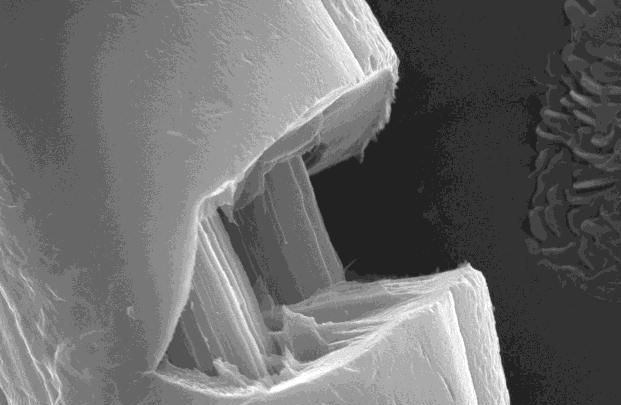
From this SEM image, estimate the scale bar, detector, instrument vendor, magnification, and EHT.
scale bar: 2000 nm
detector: SEI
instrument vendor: JEOL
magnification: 7.5 K X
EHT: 20 kV